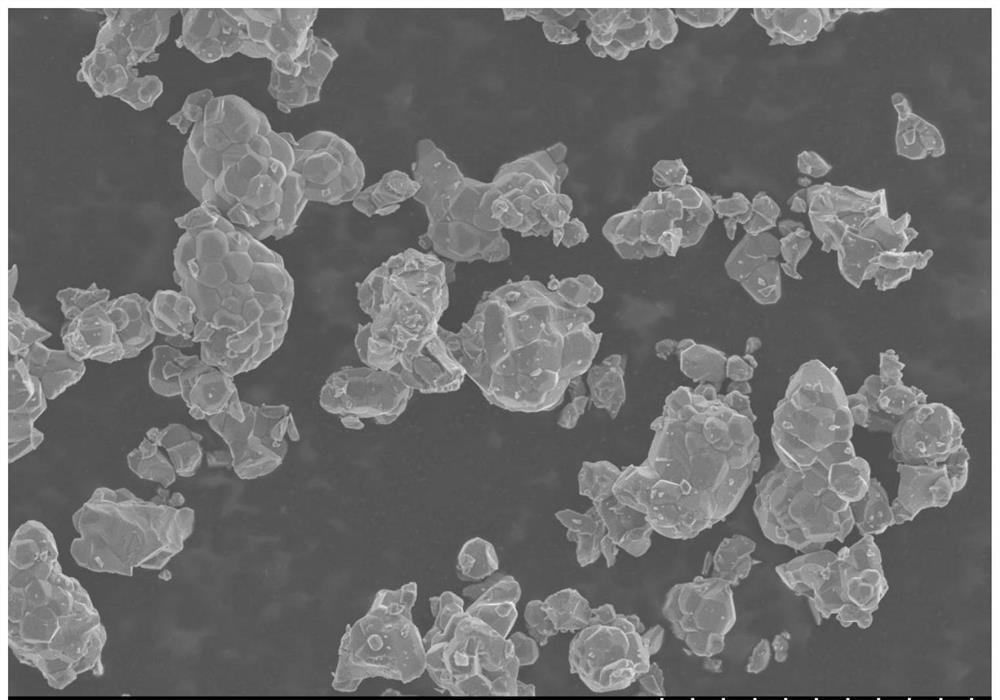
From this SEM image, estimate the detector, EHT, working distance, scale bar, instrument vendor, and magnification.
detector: SE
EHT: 5 kV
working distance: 8.2 mm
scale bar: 20000 nm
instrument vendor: Hitachi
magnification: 2 K X